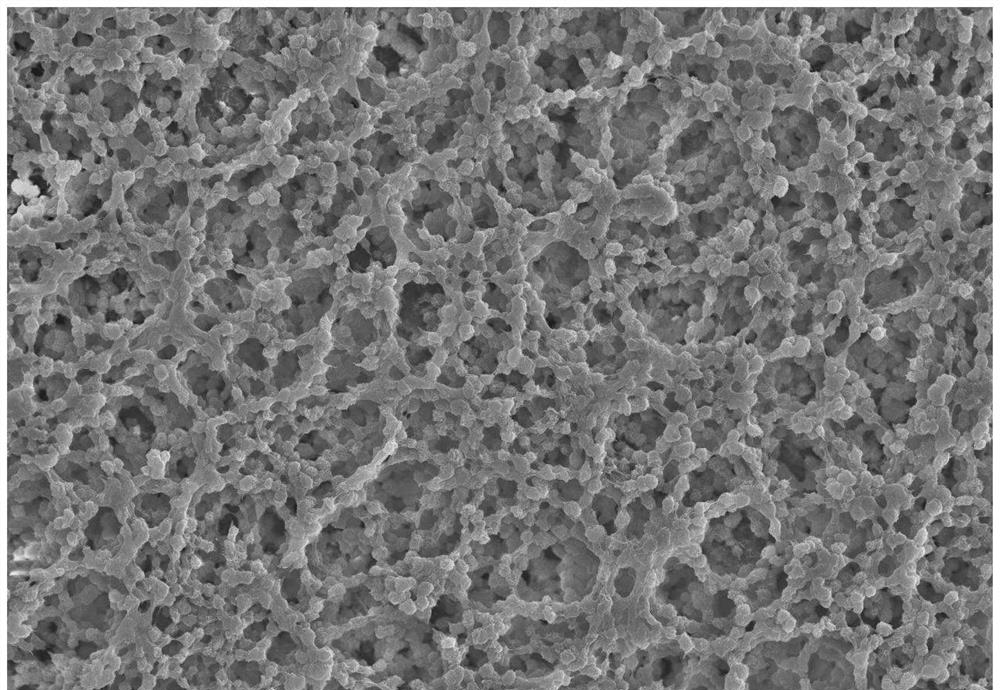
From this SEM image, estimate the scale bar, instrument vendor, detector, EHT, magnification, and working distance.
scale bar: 20000 nm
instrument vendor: Hitachi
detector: S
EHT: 20 kV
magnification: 0.5 K X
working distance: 7.4 mm